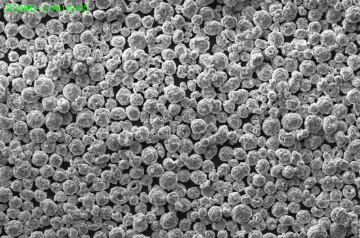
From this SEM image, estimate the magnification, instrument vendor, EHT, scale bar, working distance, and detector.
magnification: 0.3 K X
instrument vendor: Zeiss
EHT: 25 kV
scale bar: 20000 nm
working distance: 10.5 mm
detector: SE1